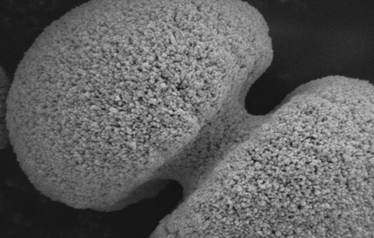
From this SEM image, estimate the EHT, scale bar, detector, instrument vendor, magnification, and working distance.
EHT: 5 kV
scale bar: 1000 nm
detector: SEI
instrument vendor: JEOL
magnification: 20 K X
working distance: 6 mm